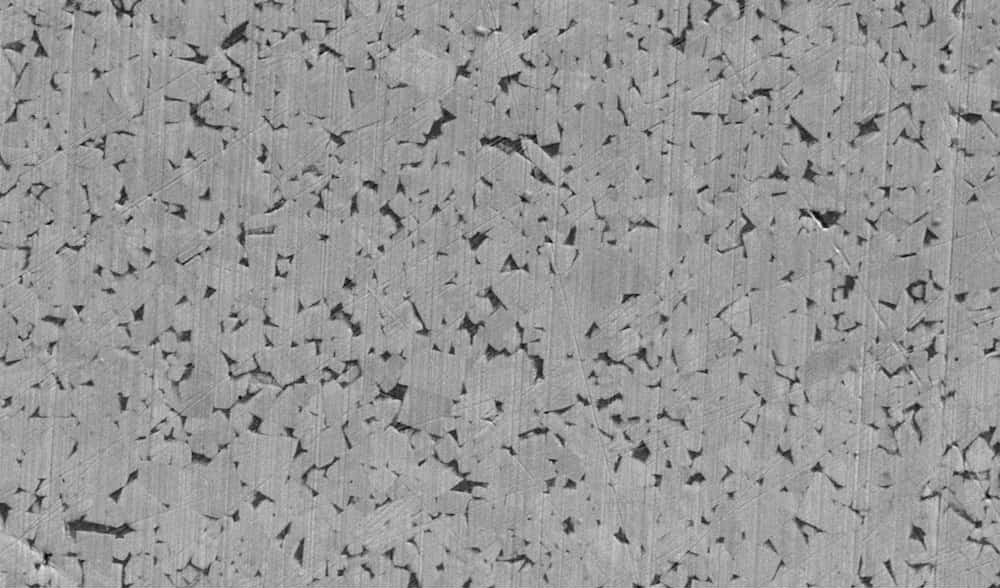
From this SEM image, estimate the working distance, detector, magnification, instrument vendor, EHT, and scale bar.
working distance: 6.9 mm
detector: SE2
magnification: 5 K X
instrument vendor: Zeiss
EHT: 5 kV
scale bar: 2000 nm